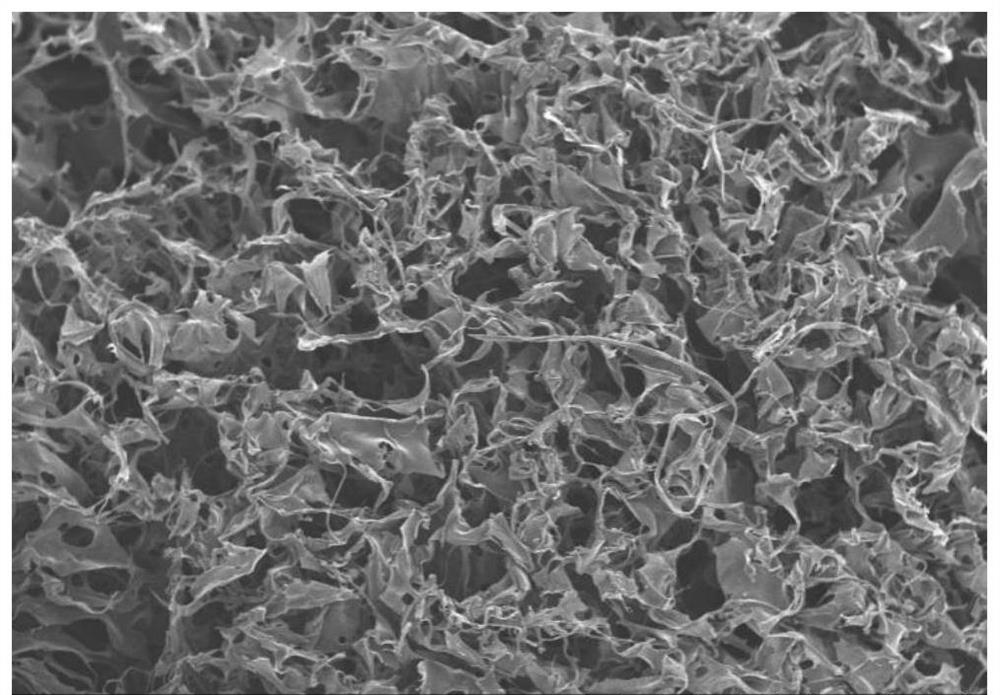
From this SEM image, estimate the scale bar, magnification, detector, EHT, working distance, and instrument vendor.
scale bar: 1000000 nm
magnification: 0.05 K X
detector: SE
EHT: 15 kV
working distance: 7.6 mm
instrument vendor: Hitachi